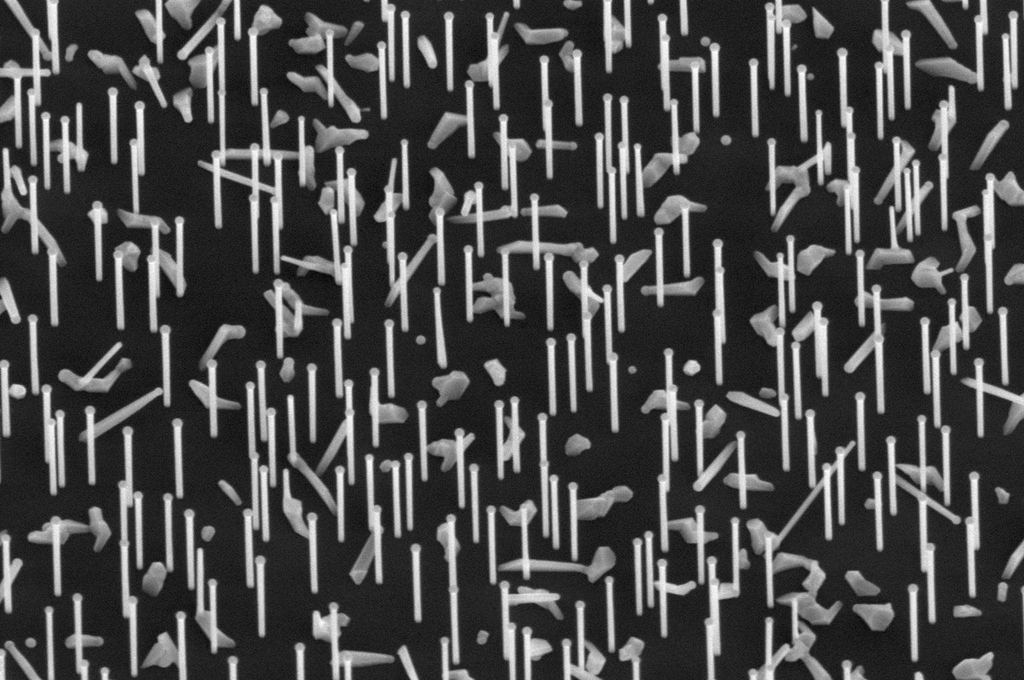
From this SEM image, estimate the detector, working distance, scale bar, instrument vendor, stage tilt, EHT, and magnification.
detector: T2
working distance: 6.9 mm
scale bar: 2000 nm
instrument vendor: FEI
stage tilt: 30°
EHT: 5 kV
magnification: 30 K X